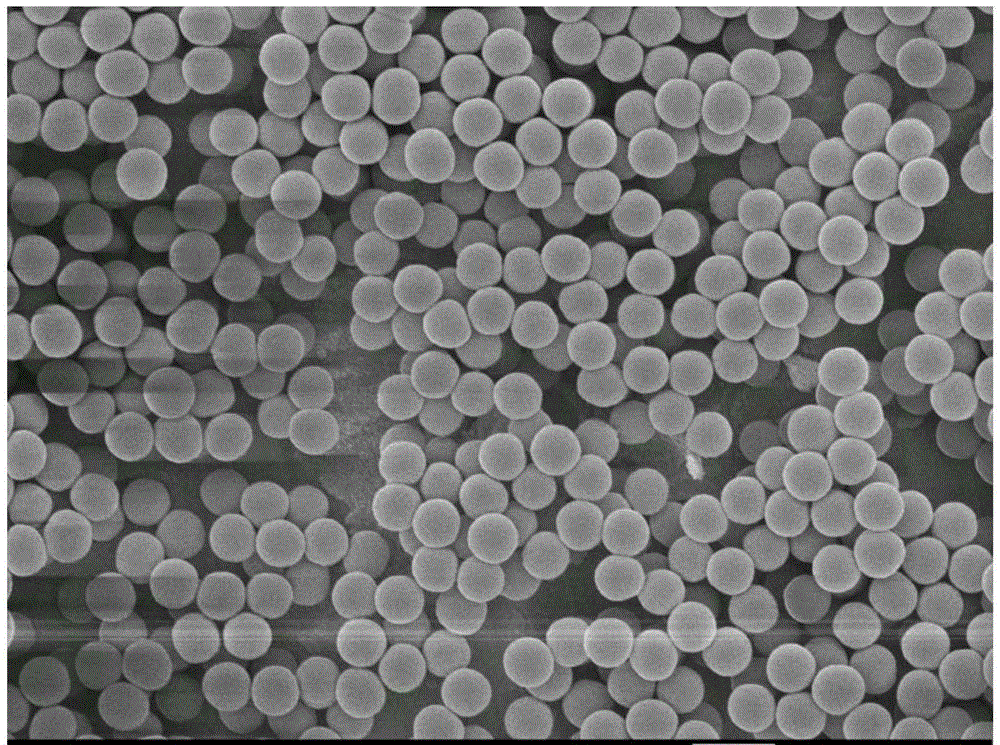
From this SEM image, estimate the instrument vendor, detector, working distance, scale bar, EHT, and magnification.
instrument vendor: JEOL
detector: SEI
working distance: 8.7 mm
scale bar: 1000 nm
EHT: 5 kV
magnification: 10 K X